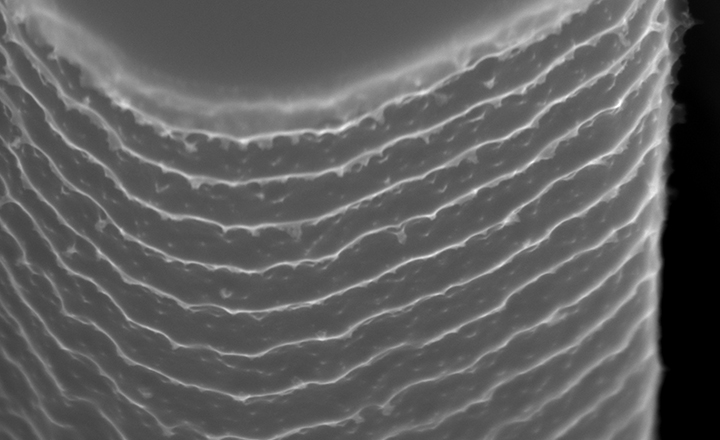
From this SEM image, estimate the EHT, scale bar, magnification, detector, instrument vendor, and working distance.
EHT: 20 kV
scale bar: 500 nm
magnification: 110 K X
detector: UD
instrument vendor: Hitachi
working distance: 8.6 mm